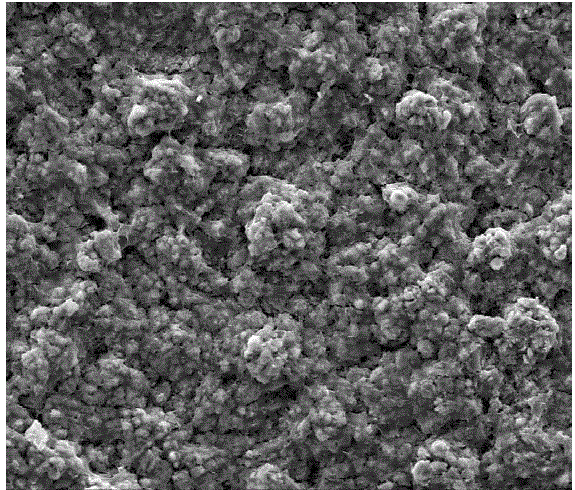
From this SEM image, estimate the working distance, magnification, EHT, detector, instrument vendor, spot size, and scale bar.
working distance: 11.7 mm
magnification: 1 K X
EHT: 20 kV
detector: ETD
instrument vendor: FEI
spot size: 5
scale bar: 100000 nm